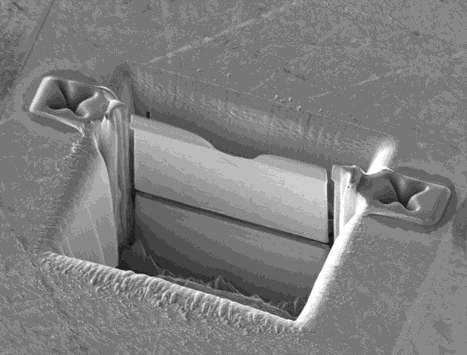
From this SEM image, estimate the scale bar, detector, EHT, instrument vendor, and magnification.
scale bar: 5000 nm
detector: CDM-E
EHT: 30 kV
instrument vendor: FEI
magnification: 10 K X